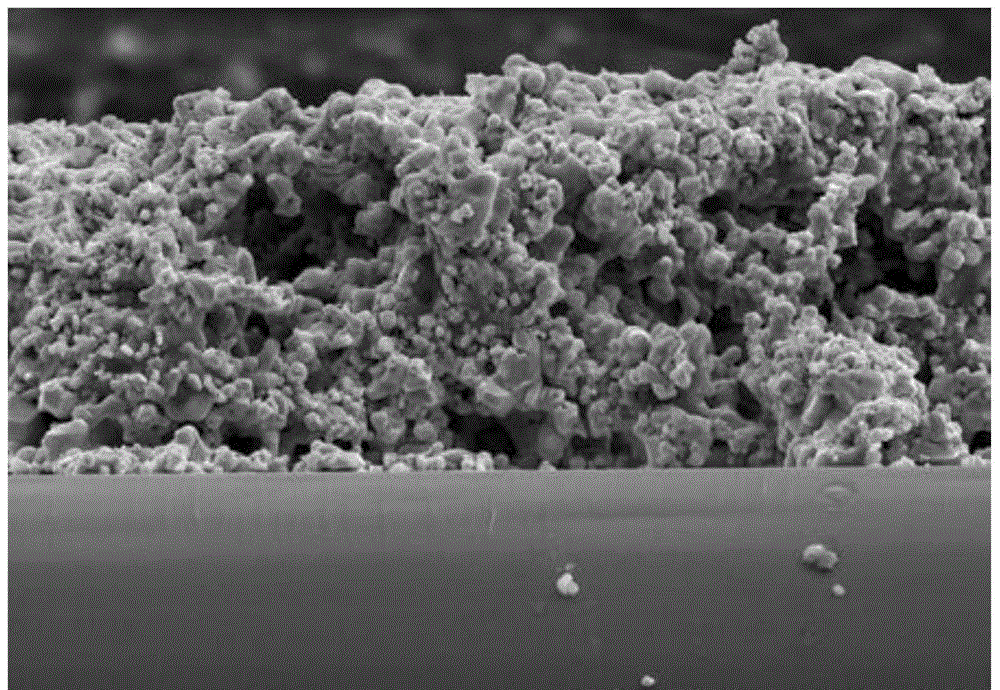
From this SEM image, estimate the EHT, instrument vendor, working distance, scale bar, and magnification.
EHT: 15 kV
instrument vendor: Hitachi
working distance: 11.4 mm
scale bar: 50000 nm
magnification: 1 K X